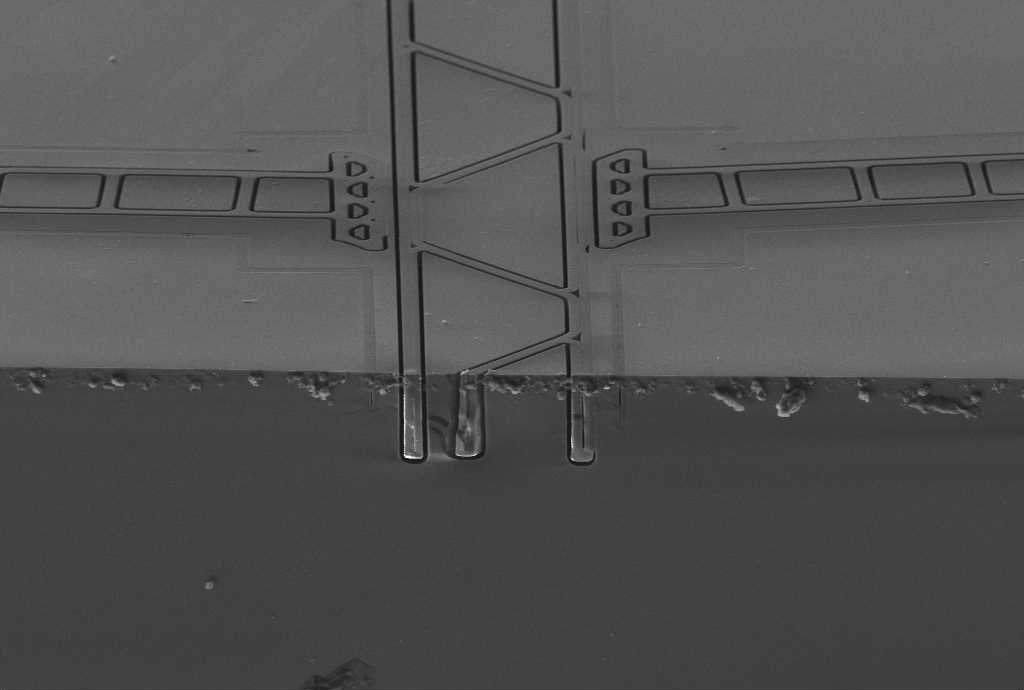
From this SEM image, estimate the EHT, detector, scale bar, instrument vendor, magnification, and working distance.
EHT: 8 kV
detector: SE2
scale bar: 10000 nm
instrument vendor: Zeiss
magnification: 0.549 K X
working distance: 7 mm